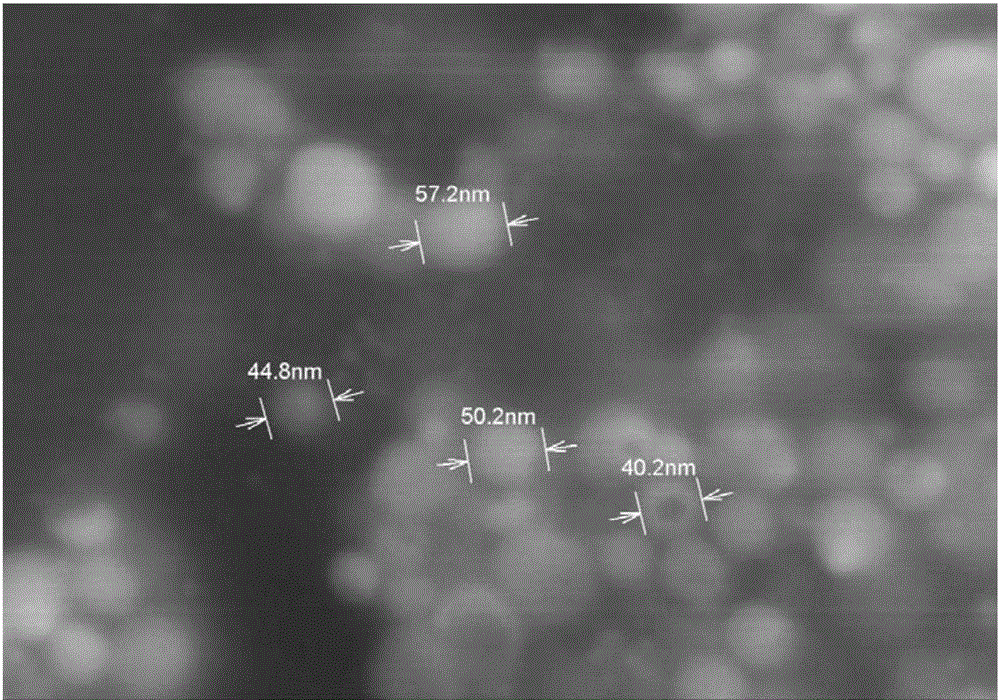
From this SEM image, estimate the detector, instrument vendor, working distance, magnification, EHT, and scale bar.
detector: SE(M)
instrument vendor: Hitachi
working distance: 7.9 mm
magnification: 200 K X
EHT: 5 kV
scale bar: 200 nm